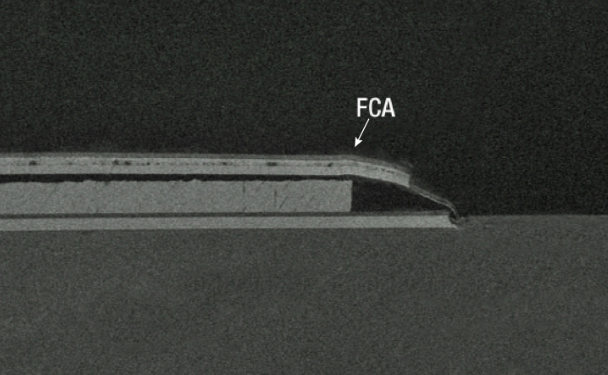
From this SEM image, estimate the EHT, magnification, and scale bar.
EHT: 3 kV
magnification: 0.187 K X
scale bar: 100000 nm